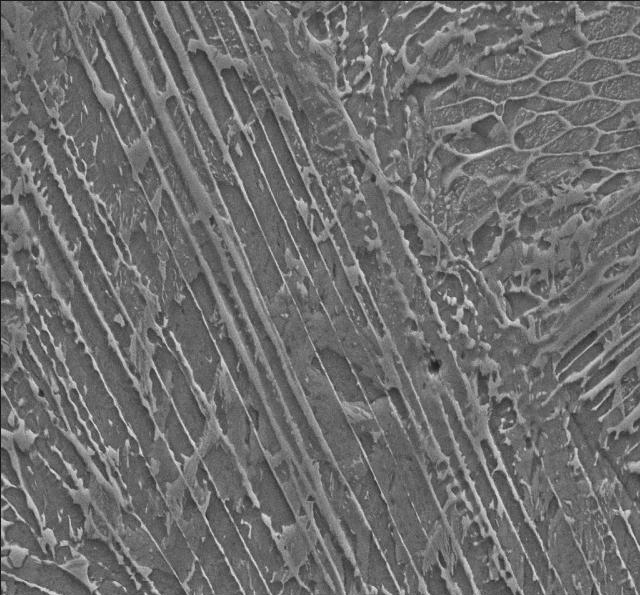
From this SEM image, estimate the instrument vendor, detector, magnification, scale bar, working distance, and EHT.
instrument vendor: Zeiss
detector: SE2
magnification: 10 K X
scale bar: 2000 nm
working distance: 8 mm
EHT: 15 kV